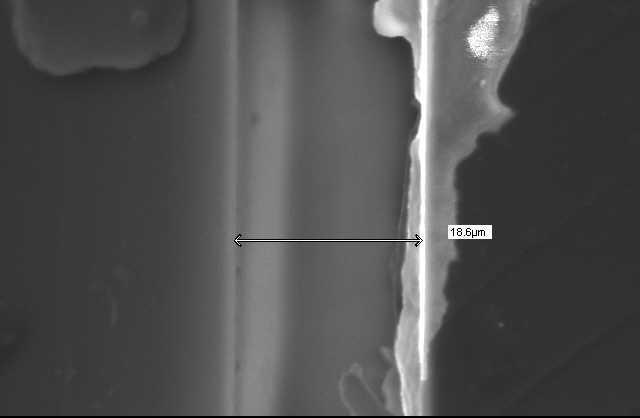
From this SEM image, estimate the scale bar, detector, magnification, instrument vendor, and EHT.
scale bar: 10000 nm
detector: SEI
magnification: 2 K X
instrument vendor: JEOL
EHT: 15 kV